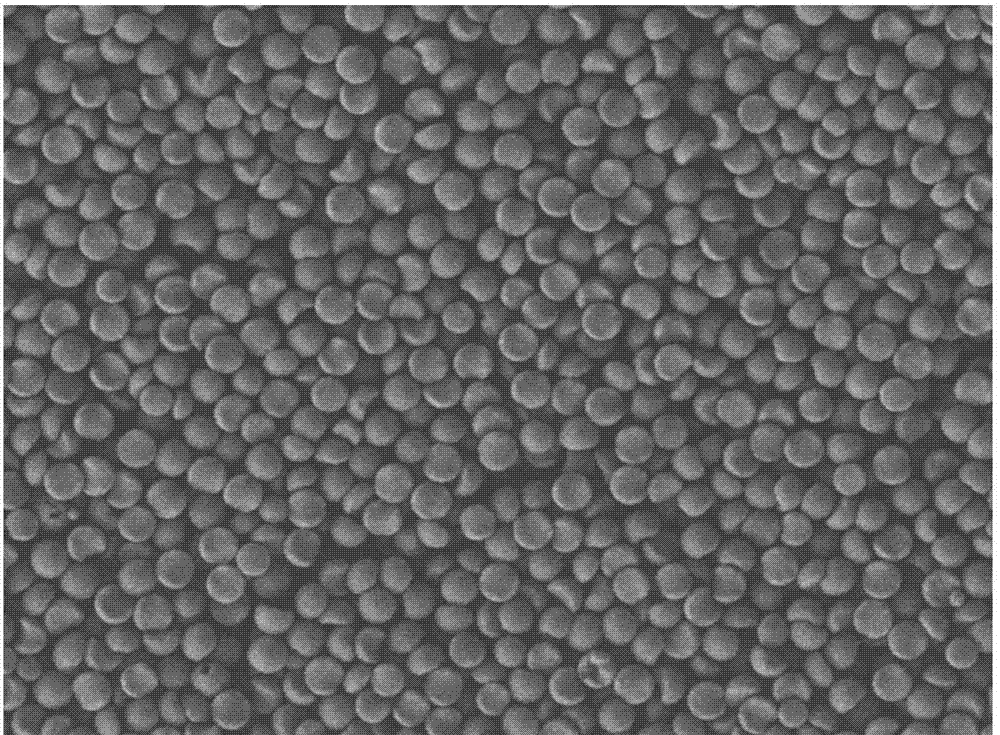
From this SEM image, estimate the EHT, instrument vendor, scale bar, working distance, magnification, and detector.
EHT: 5 kV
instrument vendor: JEOL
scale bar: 10000 nm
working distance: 7.4 mm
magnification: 2 K X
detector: LEI